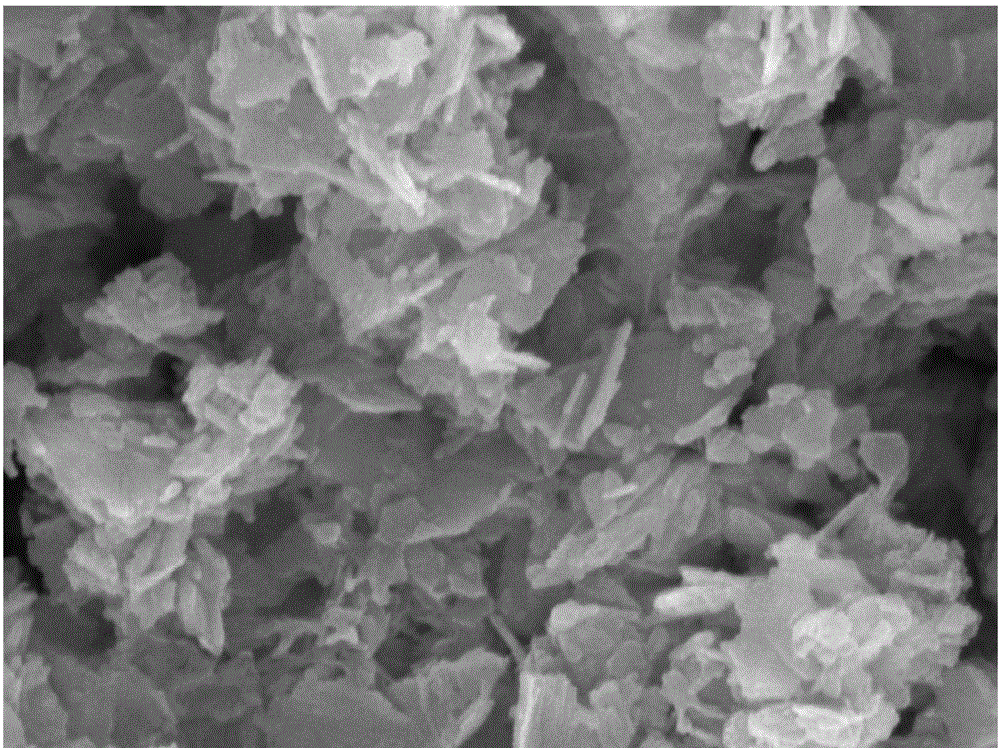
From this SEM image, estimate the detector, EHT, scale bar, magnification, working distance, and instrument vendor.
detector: SE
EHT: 20 kV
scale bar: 1000 nm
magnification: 80 K X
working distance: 14.63 mm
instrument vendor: Tescan